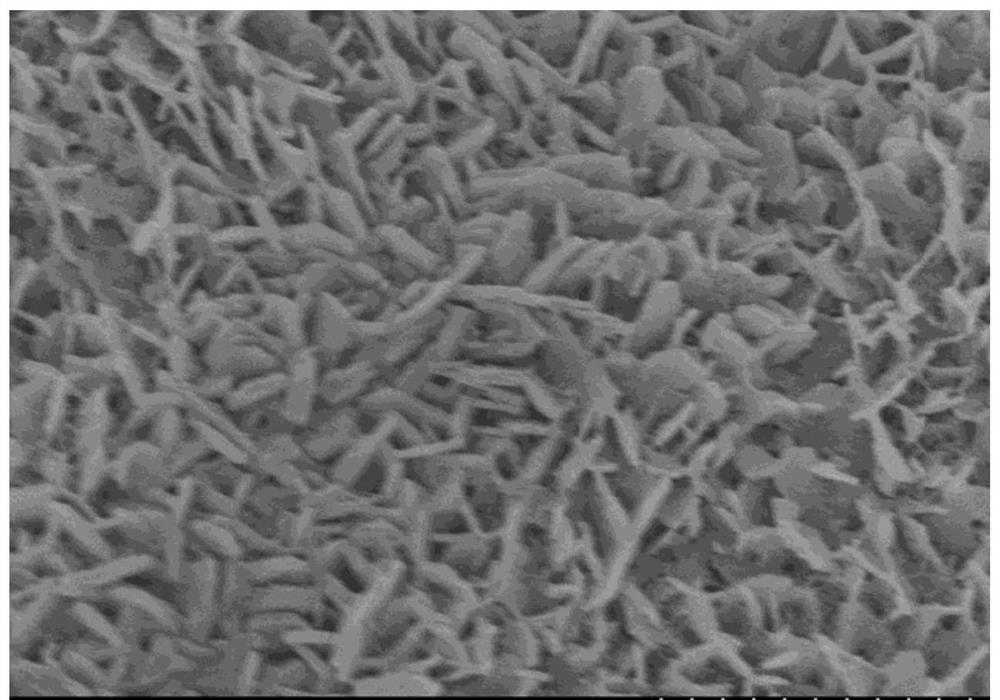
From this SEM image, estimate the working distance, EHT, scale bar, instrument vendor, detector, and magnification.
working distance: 9.5 mm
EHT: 5 kV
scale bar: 2000 nm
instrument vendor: Hitachi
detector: SE(M)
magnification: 20 K X